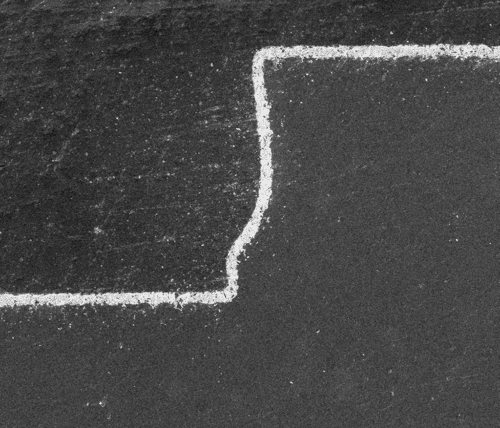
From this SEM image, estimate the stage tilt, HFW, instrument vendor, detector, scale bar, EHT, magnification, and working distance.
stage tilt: -0°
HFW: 1140 µm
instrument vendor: FEI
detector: ETD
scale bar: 300000 nm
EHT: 5 kV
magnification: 0.2 K X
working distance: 9.9 mm